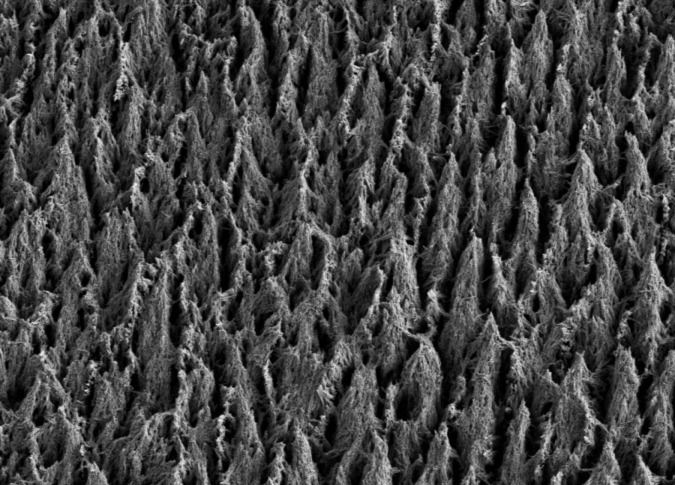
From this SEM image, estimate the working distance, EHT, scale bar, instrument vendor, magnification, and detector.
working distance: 6.4 mm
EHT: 2 kV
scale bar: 1000 nm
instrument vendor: Zeiss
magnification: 4.96 K X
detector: SE2